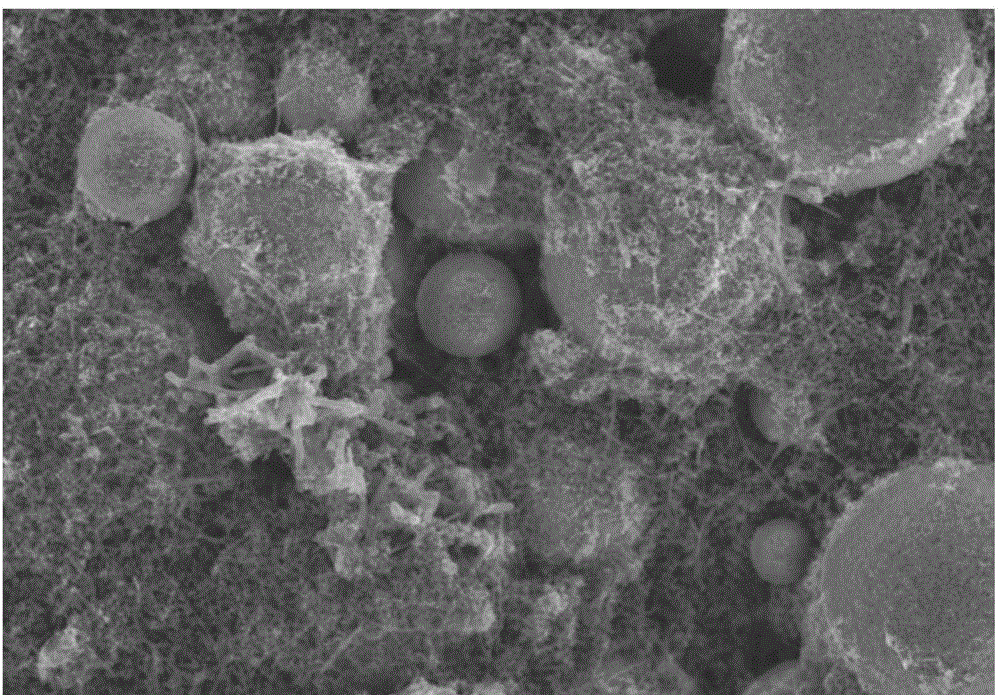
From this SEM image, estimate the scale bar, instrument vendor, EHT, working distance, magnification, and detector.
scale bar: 20000 nm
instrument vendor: Hitachi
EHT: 10 kV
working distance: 8 mm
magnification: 2 K X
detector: SE(M)